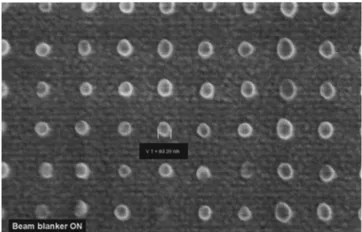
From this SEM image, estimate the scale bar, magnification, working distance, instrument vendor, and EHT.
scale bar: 200 nm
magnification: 50 K X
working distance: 3 mm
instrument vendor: Zeiss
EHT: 3 kV